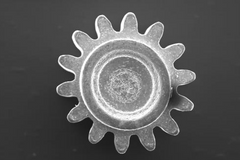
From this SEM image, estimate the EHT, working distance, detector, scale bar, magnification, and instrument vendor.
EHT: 20 kV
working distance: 8.5 mm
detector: SE1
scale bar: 200000 nm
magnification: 0.05 K X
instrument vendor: Zeiss